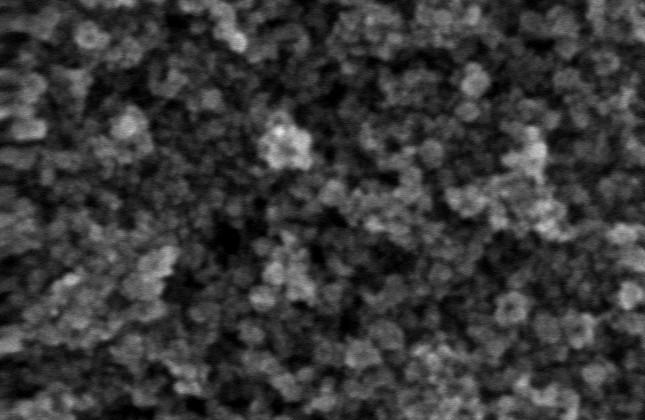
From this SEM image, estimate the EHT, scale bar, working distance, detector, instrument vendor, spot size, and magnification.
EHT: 7 kV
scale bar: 200 nm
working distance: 3.9 mm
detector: TLD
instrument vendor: FEI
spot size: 2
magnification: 150 K X